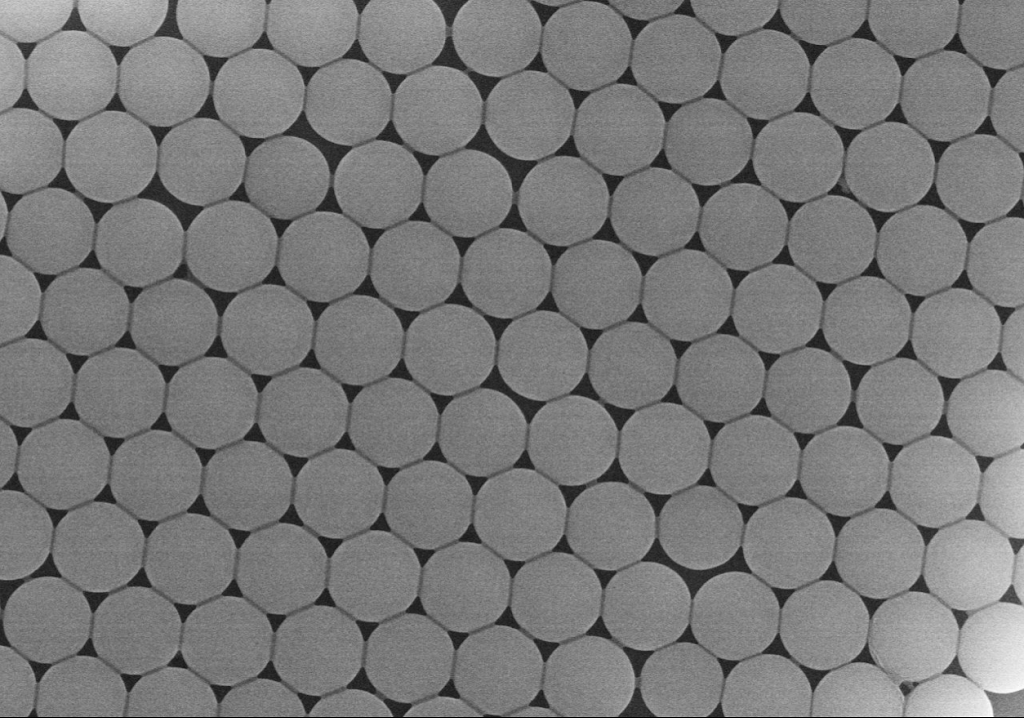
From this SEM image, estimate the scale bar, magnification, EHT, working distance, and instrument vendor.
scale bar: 5000 nm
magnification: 10 K X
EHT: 15 kV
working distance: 15.1 mm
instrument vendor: Hitachi